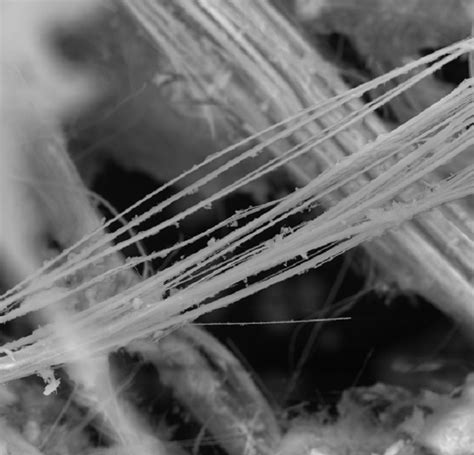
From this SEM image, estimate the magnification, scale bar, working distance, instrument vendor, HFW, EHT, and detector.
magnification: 1.8 K X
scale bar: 20000 nm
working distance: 10.2 mm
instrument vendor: Tescan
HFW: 154 µm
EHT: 20 kV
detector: SE